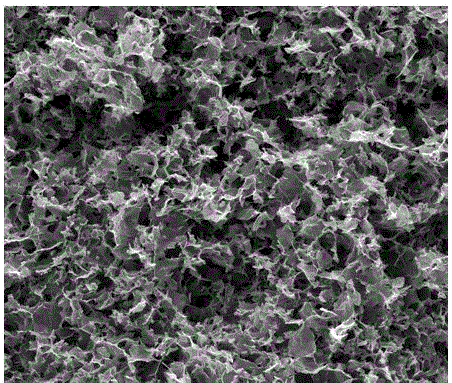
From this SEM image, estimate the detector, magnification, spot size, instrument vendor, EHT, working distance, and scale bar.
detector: ETD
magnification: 1.2 K X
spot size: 4.5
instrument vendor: FEI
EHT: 15 kV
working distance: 9 mm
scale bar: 50000 nm